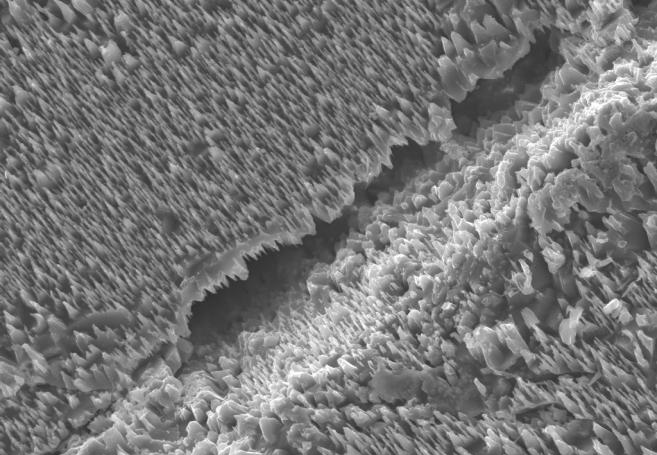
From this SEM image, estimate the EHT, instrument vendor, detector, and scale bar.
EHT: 20 kV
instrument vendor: Tescan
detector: SE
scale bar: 50000 nm